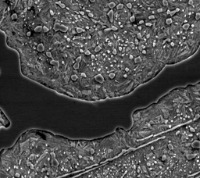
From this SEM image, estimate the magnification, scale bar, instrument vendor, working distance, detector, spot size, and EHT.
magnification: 1.905 K X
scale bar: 10000 nm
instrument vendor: FEI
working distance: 10 mm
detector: SE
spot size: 6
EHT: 20 kV